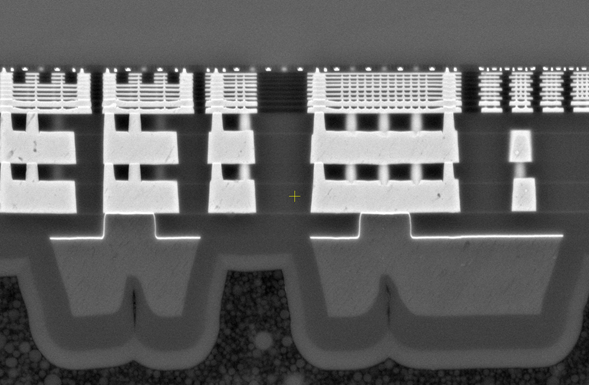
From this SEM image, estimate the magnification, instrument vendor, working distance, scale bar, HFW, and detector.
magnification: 20 K X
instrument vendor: Thermo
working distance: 2.7 mm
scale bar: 10000 nm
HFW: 20.7 µm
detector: T1